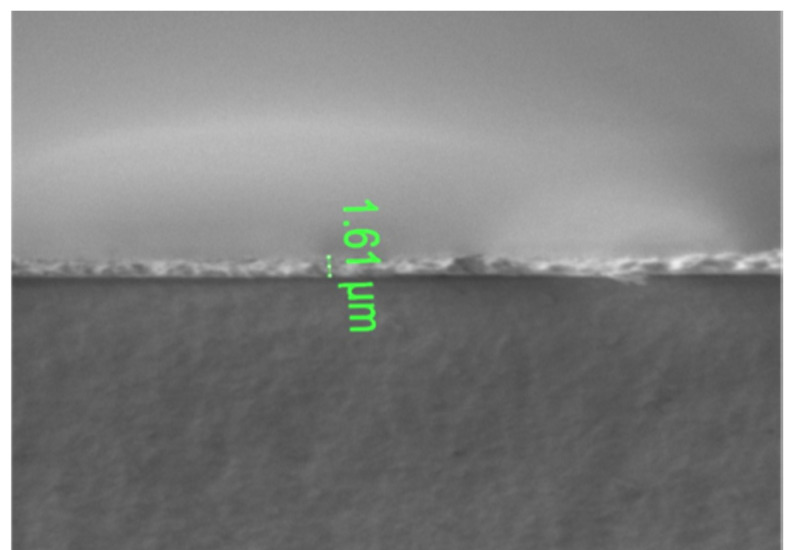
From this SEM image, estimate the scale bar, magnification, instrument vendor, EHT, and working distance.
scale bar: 40000 nm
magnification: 1.5 K X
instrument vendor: FEI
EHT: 1 kV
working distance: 14.4 mm